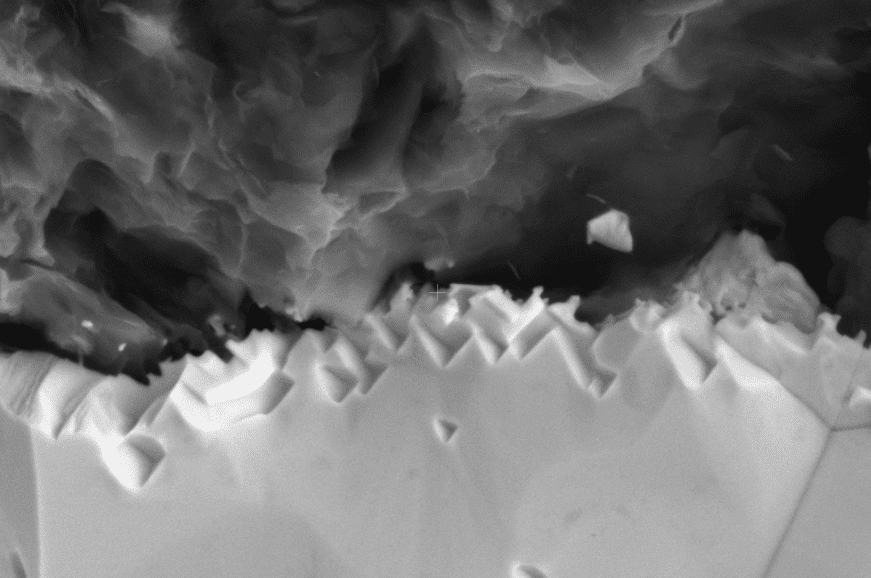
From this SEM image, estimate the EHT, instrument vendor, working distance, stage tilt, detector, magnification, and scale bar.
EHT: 10 kV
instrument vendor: FEI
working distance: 10.6 mm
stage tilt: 0°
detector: ETD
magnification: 10 K X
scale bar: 3000 nm